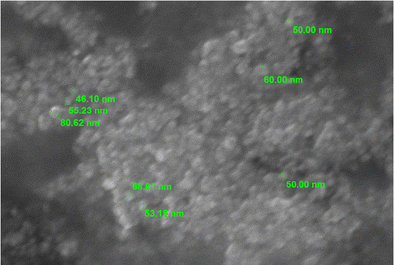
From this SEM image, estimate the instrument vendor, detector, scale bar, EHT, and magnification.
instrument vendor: JEOL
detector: SEI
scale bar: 500 nm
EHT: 20 kV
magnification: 40 K X